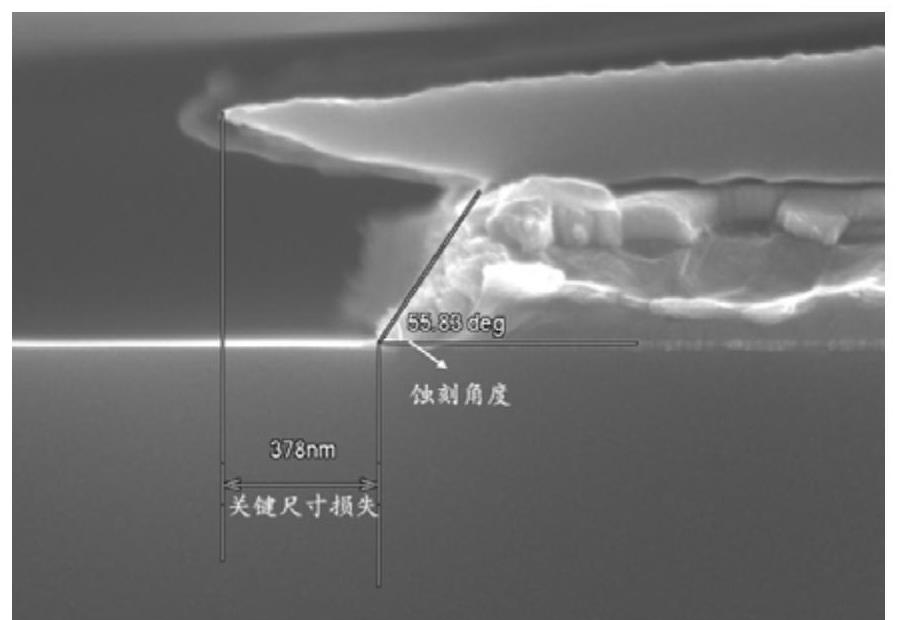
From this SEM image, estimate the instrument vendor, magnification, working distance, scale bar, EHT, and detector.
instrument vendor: Hitachi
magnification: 60 K X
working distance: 10.5 mm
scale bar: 500 nm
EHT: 10 kV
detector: SE(U)